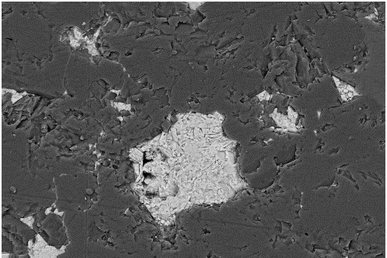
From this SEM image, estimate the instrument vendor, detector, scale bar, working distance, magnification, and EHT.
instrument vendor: Zeiss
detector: AsB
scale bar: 10000 nm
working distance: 5.9 mm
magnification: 1.79 K X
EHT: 10 kV